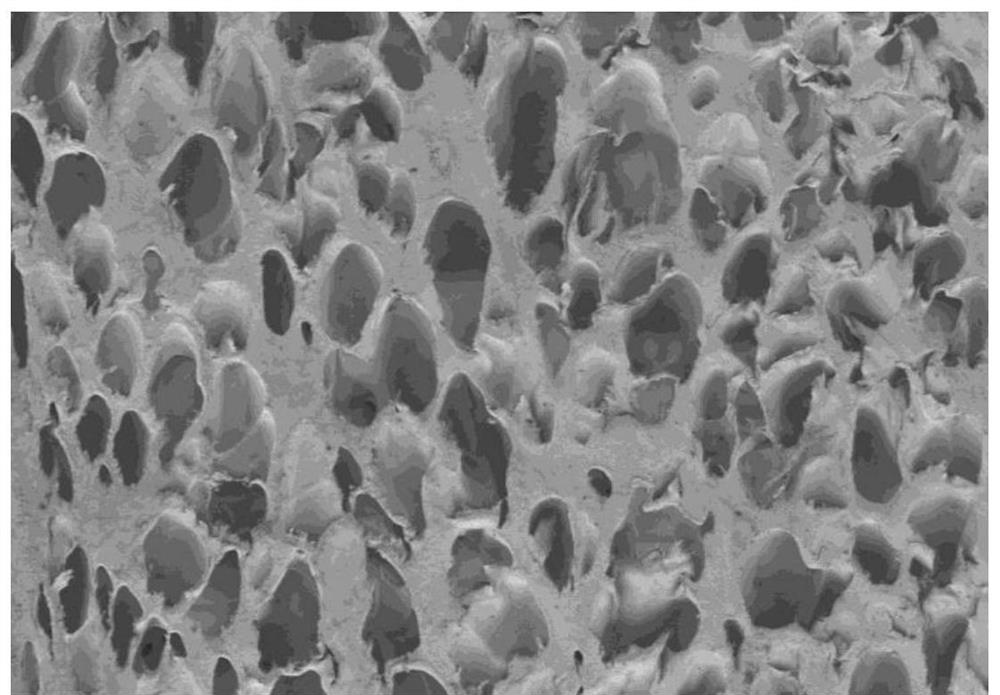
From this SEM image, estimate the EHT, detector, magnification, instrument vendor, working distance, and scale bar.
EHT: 1 kV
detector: SE(M)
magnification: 0.03 K X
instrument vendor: Hitachi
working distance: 9.9 mm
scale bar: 1000000 nm